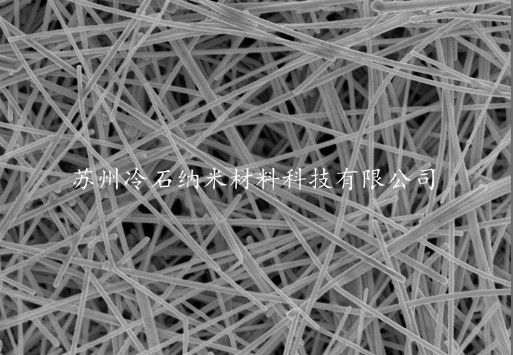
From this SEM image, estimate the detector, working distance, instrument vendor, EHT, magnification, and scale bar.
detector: SE(M)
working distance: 8.3 mm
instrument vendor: Hitachi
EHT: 5 kV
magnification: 20 K X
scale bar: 2000 nm